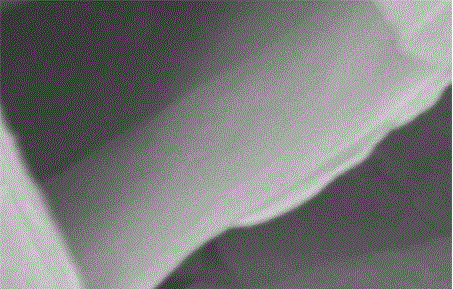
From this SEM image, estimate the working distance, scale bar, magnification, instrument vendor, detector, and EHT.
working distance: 8.2 mm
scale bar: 500 nm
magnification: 100 K X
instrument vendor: Hitachi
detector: SE(M)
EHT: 5 kV